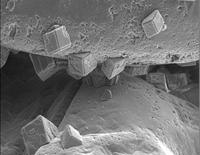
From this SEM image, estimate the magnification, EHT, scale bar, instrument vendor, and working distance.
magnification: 1.5 K X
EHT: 7 kV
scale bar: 10000 nm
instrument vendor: JEOL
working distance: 14 mm